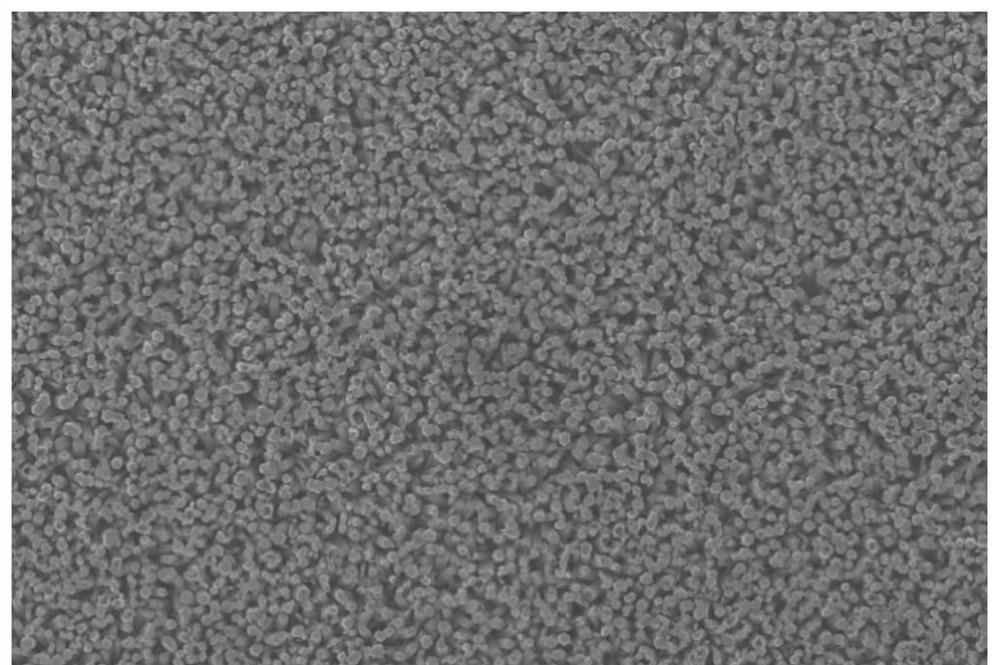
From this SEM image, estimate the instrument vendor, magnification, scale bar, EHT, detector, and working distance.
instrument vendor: Zeiss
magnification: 2 K X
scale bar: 2000 nm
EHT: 15 kV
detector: InLens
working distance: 5.2 mm